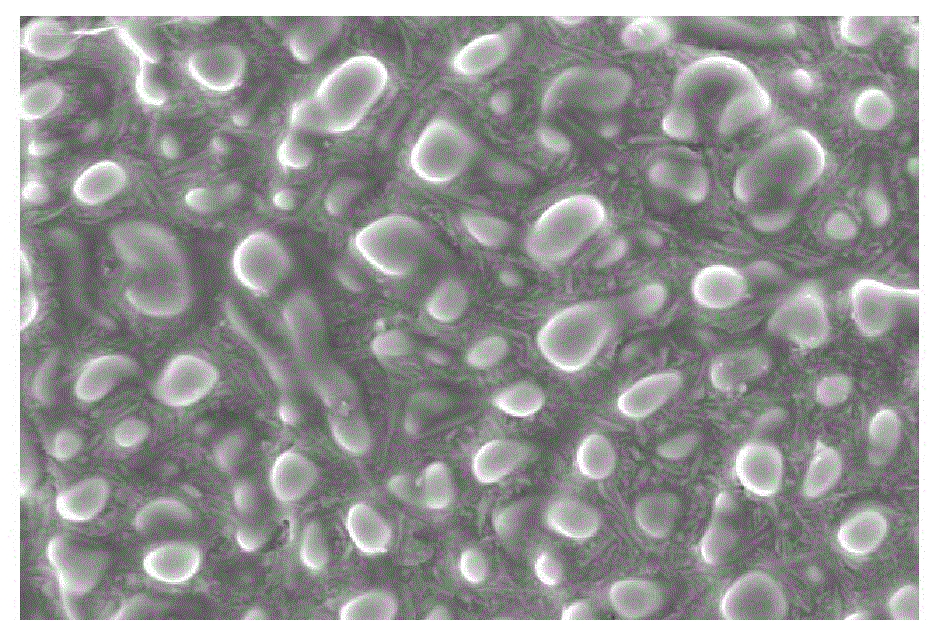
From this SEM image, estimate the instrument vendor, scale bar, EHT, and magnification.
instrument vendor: JEOL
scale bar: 10000 nm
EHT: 20 kV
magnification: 1 K X